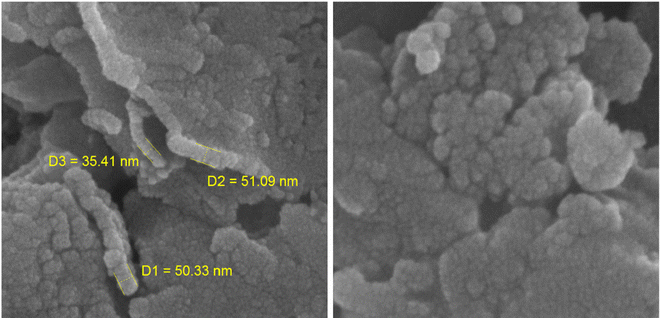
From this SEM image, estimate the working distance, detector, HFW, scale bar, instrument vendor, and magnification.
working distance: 5.05 mm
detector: InBeam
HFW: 1.04 µm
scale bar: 200 nm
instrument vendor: Tescan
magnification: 200 K X